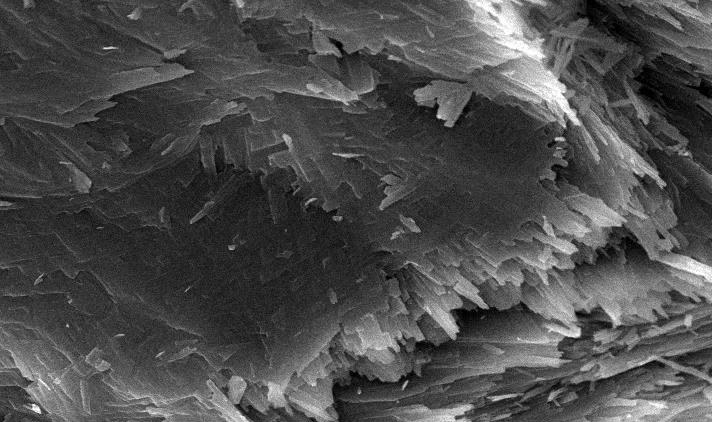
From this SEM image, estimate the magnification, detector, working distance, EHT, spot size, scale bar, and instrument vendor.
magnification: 10 K X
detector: SE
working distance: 12.1 mm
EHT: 20 kV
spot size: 3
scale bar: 5000 nm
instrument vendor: FEI